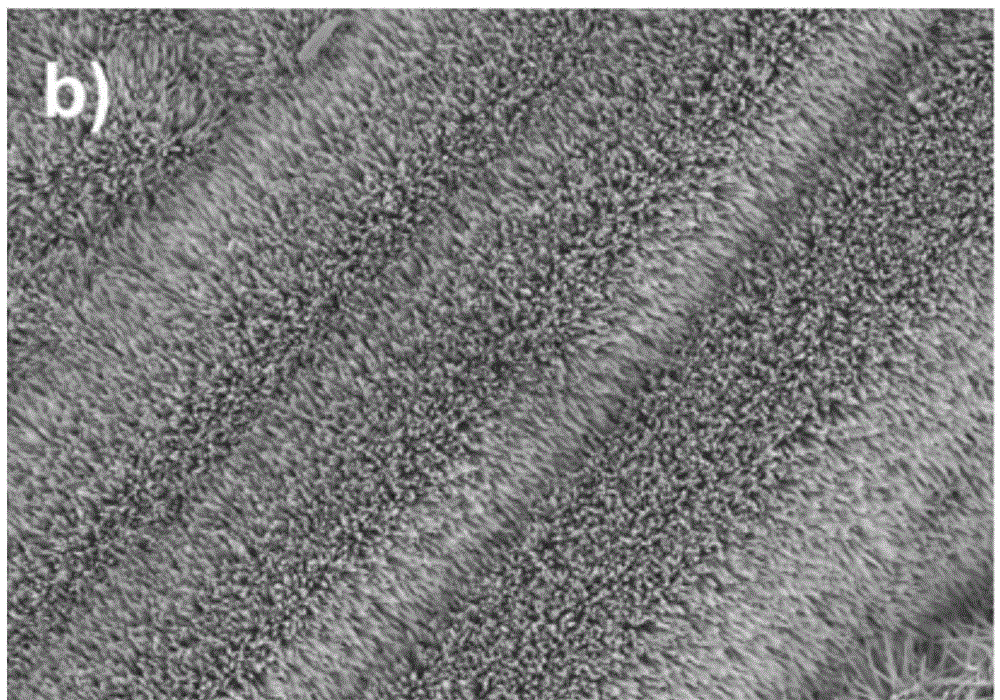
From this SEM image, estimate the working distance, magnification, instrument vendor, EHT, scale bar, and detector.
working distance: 9.7 mm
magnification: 5 K X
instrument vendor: Hitachi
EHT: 10 kV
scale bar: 10000 nm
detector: SE(M)